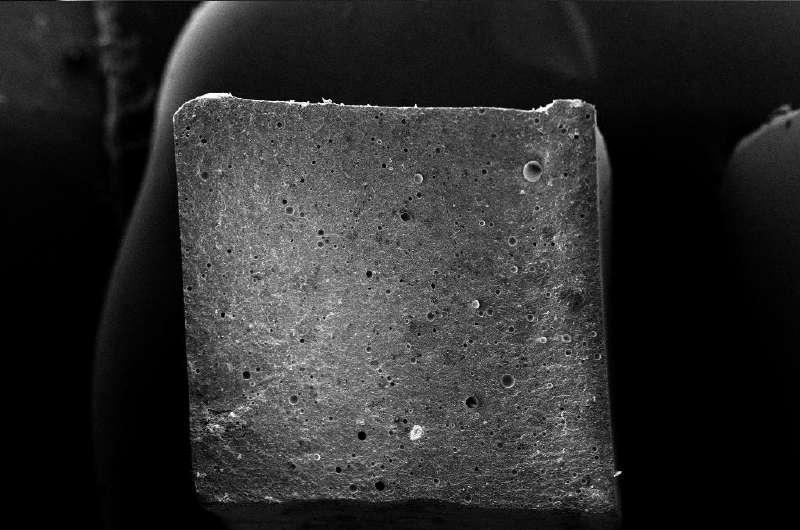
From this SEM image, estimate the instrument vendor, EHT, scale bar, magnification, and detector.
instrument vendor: Zeiss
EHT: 20 kV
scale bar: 1000000 nm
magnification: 0.085 K X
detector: SE1